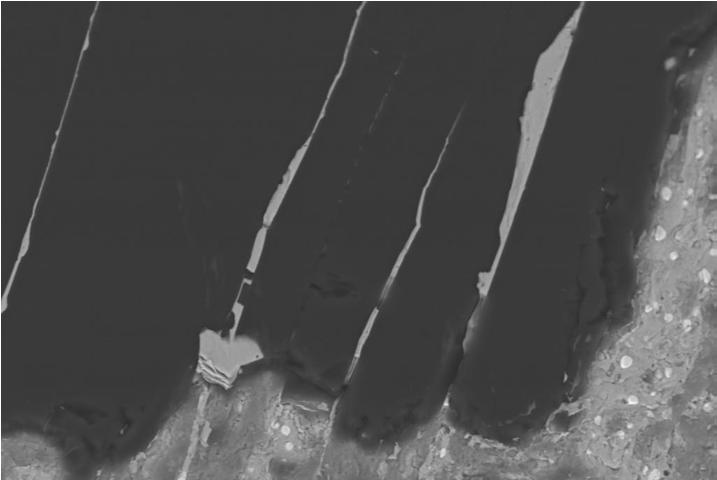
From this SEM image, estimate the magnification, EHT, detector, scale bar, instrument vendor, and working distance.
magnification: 4 K X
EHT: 15 kV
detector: CZ BSD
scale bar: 10000 nm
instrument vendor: Zeiss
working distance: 6.5 mm